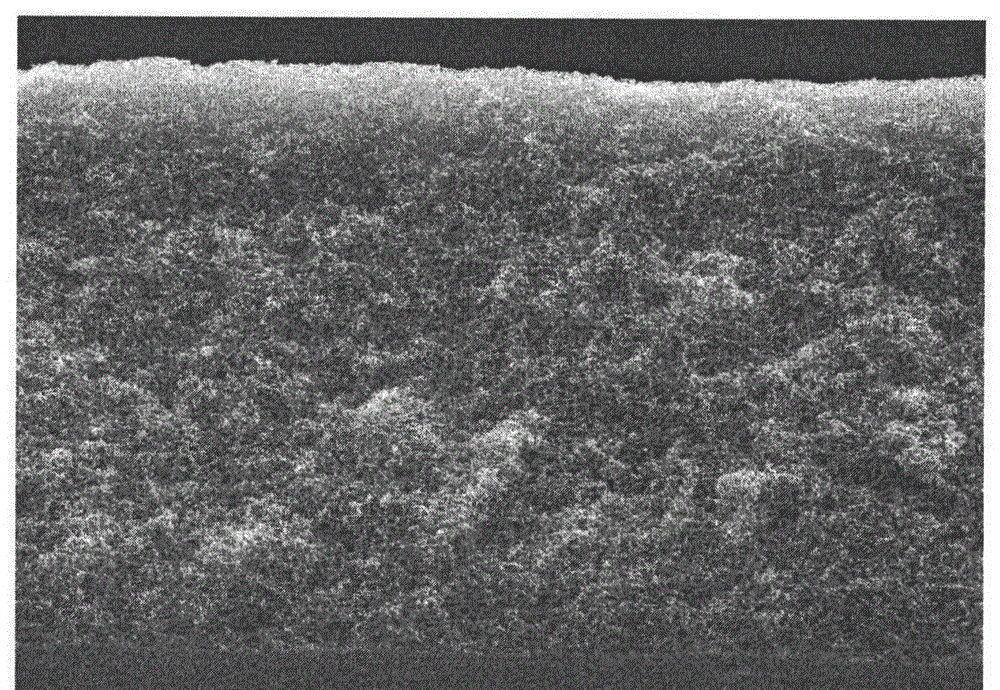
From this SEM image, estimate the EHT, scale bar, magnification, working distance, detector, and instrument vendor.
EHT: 20 kV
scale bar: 50000 nm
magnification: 0.8 K X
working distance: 10.5 mm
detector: SE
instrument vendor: Hitachi